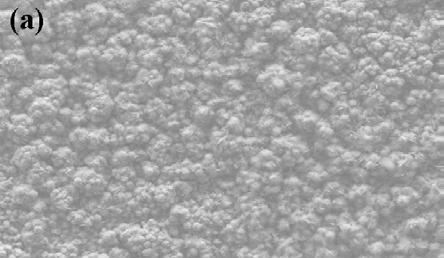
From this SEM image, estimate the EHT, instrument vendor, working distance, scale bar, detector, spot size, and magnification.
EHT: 20 kV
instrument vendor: FEI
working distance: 10.3 mm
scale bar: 20000 nm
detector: SE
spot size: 4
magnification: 1 K X